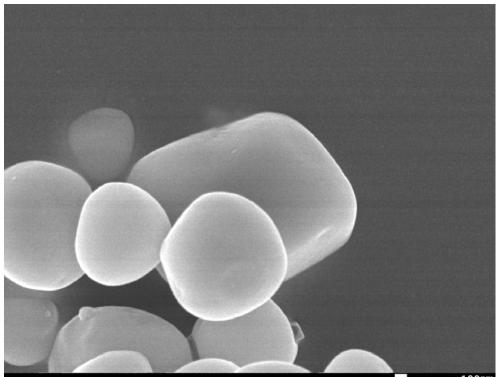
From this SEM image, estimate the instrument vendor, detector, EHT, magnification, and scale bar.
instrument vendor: JEOL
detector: SEI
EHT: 15 kV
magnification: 30 K X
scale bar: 100 nm